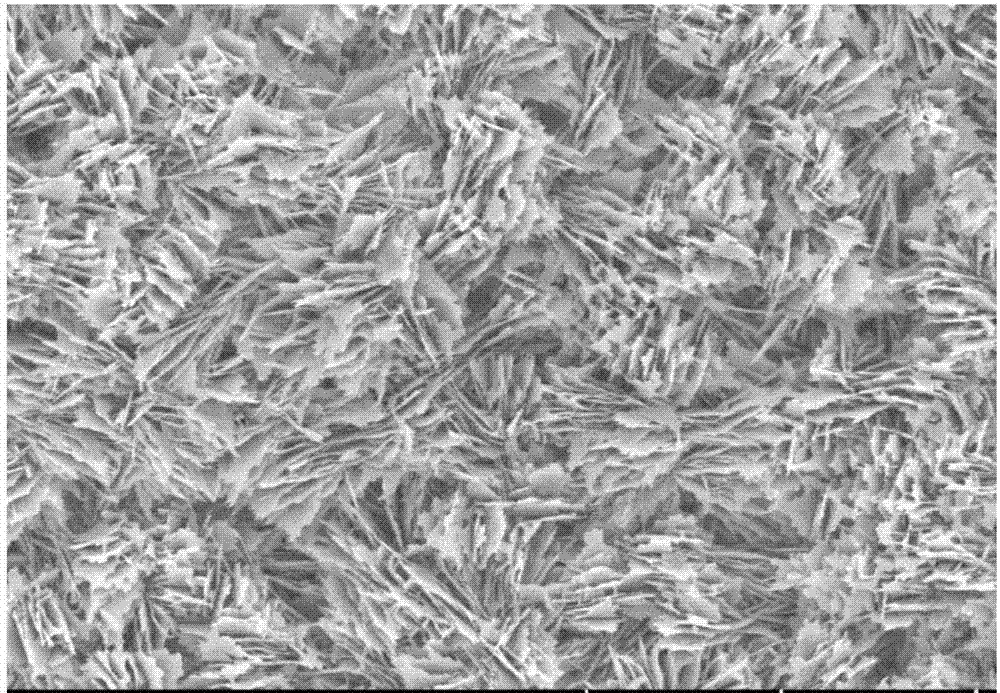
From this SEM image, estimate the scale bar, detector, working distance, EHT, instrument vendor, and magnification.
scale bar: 10000 nm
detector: SE(UL)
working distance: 8.7 mm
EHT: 5 kV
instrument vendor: Hitachi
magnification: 5 K X